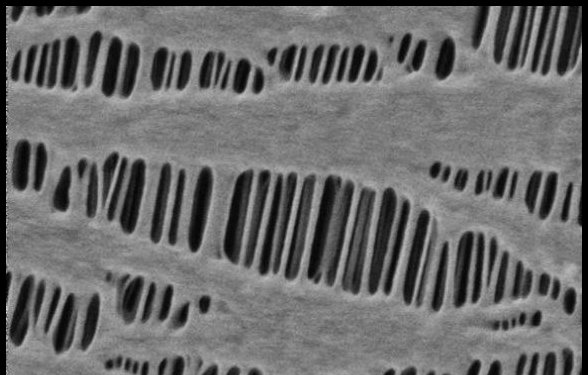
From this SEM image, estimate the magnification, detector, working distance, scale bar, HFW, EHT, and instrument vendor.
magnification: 50 K X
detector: T1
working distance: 3.1 mm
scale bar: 1000 nm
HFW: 2.54 µm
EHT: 0.3 kV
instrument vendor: Thermo